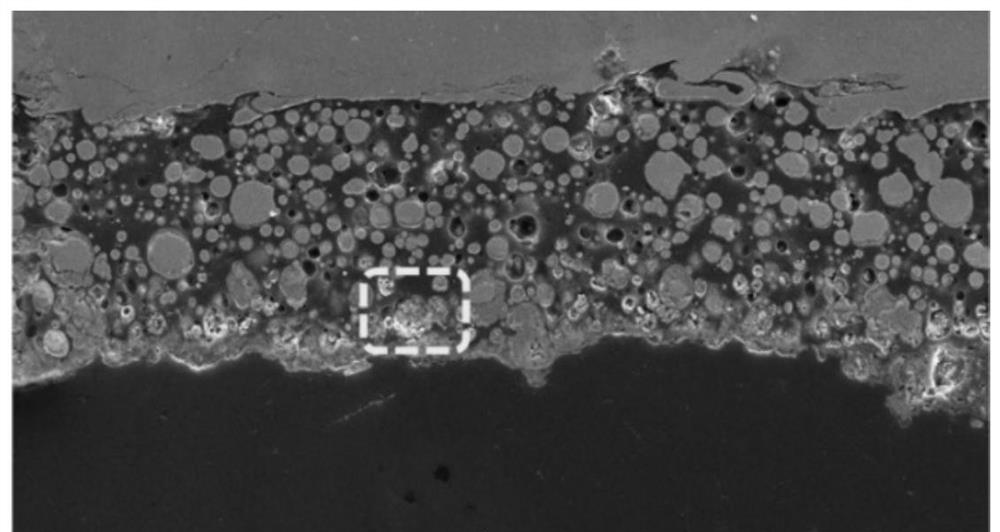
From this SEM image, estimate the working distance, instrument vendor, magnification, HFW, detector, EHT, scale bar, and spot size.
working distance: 11.1714 mm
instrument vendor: FEI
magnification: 1 K X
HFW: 414 µm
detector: ETD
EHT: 20 kV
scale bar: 100000 nm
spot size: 3